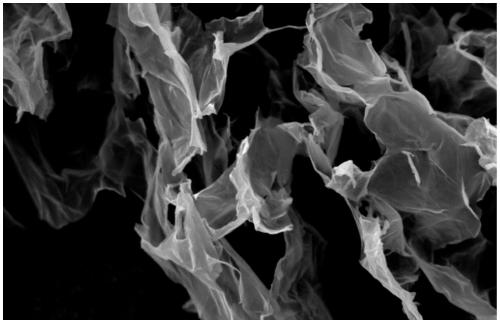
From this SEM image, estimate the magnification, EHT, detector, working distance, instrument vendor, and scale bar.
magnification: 5 K X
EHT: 5 kV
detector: SE1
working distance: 8 mm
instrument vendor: Zeiss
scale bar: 1000 nm